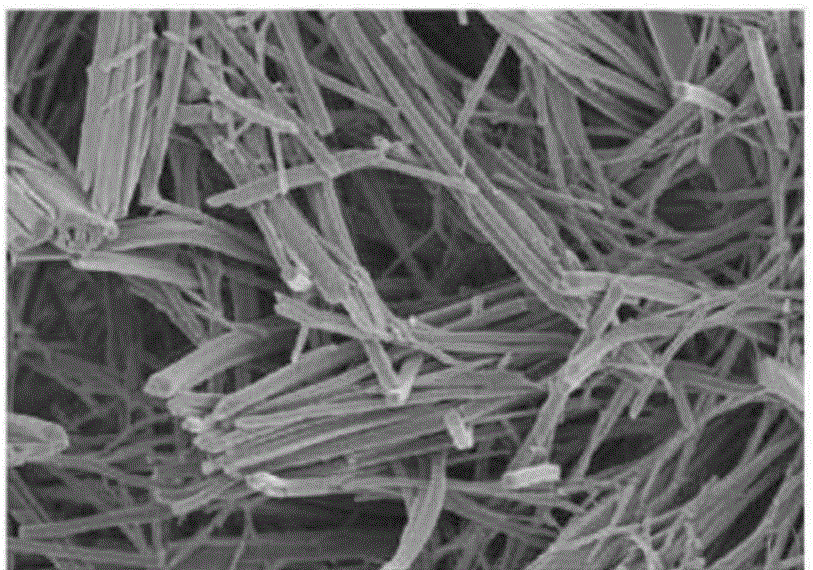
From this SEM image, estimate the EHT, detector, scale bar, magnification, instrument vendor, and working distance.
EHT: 3 kV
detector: SE(M)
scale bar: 5000 nm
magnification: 6 K X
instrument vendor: Hitachi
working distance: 8.9 mm